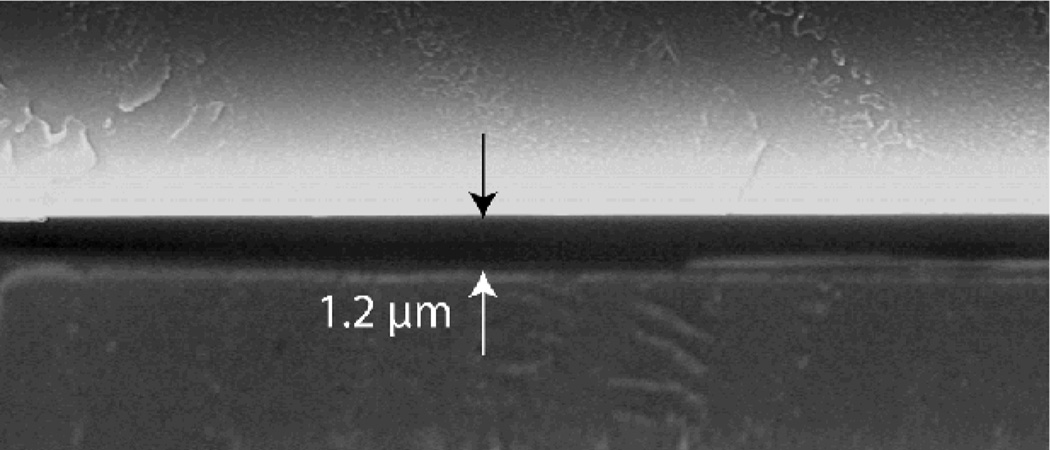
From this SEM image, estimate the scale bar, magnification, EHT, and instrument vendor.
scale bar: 10000 nm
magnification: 4.58 K X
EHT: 20 kV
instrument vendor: other